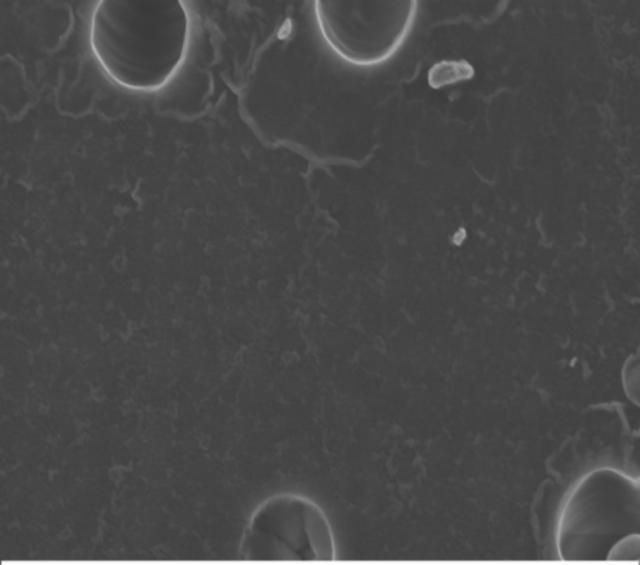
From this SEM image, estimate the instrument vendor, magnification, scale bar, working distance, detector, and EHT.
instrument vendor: Zeiss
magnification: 65 K X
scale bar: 200 nm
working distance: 3 mm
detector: InLens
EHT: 3 kV